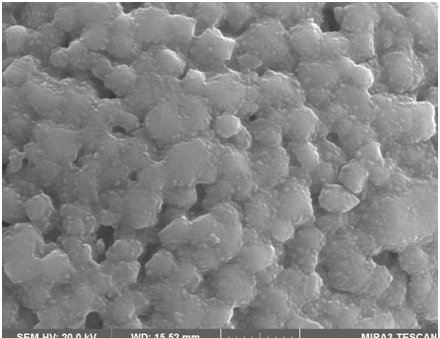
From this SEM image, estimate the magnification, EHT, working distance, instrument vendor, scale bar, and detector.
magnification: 50 K X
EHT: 20 kV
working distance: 15.52 mm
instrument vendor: Tescan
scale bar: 1000 nm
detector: SE